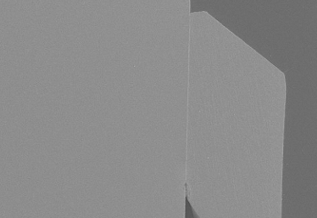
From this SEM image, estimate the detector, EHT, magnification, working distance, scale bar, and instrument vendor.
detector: SE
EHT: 10 kV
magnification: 0.05 K X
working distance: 15.1 mm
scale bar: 1000000 nm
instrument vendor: JEOL